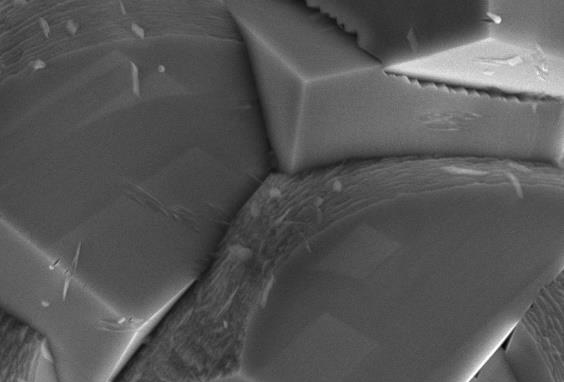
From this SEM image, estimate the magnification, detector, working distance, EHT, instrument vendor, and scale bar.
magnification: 5 K X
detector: SEI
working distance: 10 mm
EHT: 15 kV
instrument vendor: JEOL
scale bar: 5000 nm